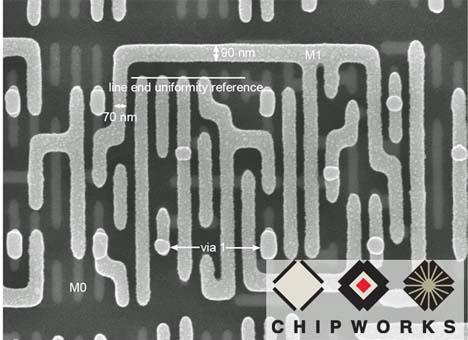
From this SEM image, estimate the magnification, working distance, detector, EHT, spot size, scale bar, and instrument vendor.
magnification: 50 K X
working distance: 5.5 mm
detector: TLD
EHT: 10 kV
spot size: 3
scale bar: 500 nm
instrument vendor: FEI